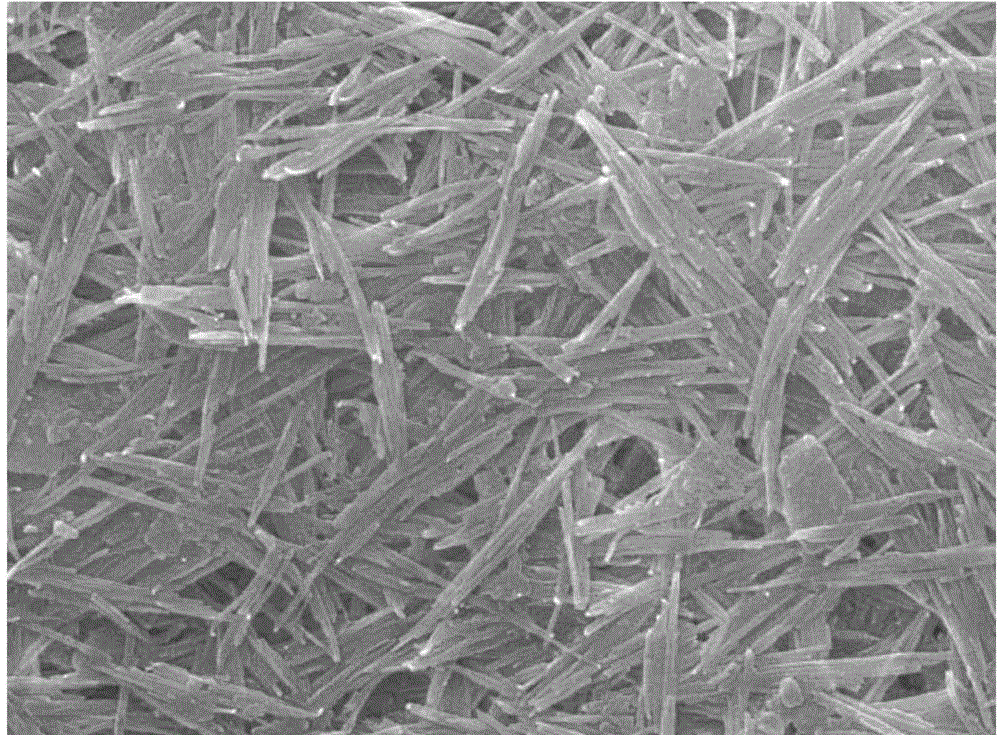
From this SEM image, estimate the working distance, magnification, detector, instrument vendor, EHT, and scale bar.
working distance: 12.4 mm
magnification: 20 K X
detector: SEI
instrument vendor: JEOL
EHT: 20 kV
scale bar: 1000 nm